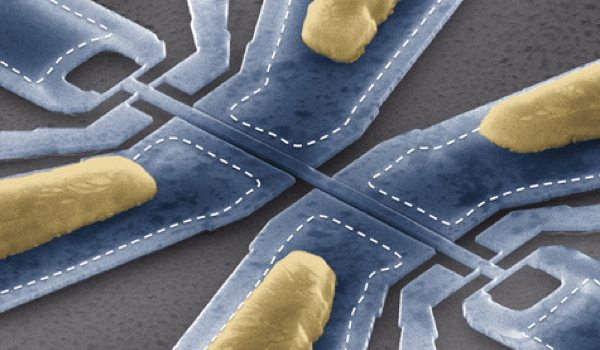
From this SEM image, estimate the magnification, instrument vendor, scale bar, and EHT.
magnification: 10 K X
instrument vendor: FEI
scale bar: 2000 nm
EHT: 15 kV